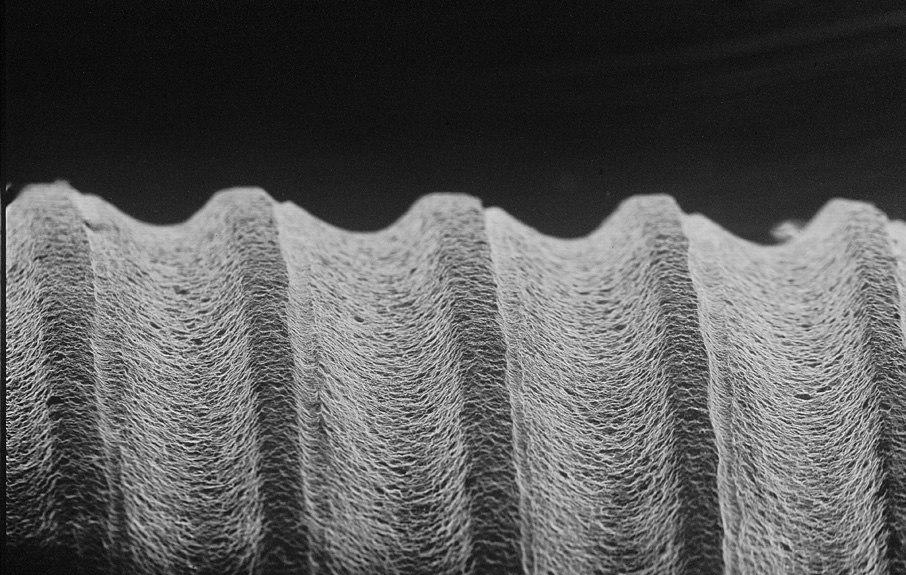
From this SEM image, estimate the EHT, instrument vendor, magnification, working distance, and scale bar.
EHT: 25 kV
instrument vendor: JEOL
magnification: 0.1 K X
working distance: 24 mm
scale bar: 100000 nm